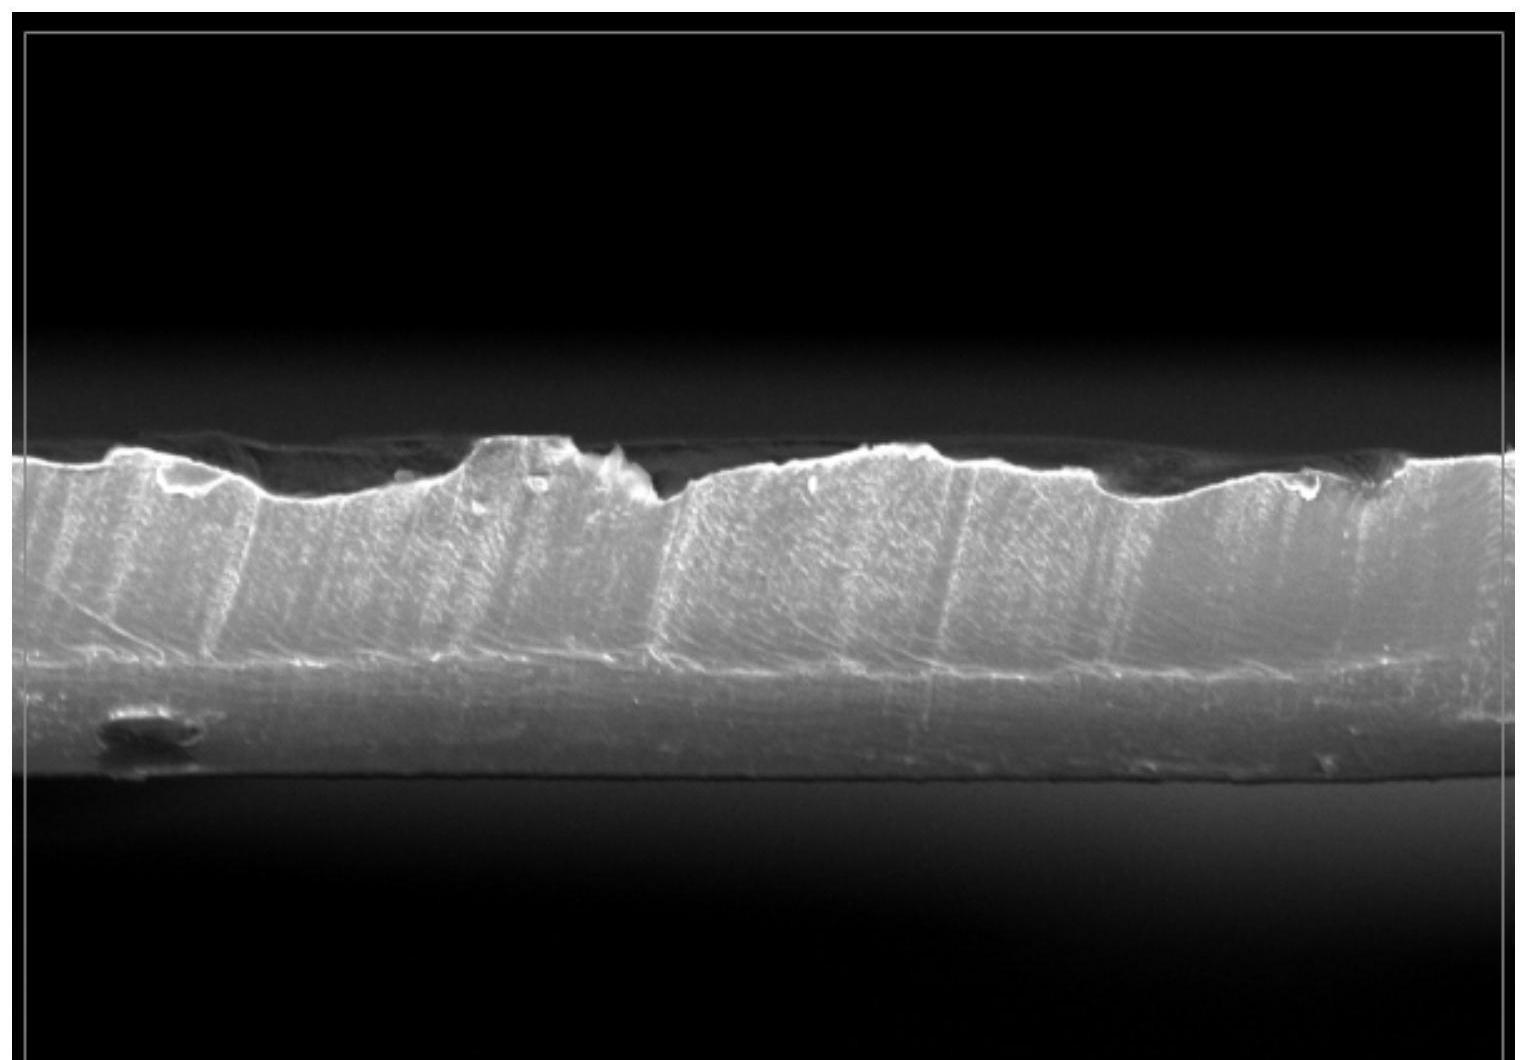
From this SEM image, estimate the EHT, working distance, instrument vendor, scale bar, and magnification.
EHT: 5 kV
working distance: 7.9 mm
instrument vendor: other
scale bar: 20000 nm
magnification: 1 K X